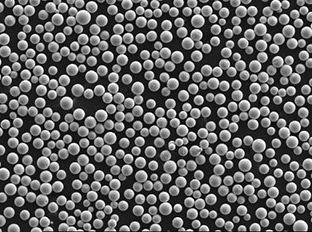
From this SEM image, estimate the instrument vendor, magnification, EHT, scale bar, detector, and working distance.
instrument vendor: JEOL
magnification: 0.1 K X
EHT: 20 kV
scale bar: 100000 nm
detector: SEI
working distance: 12 mm